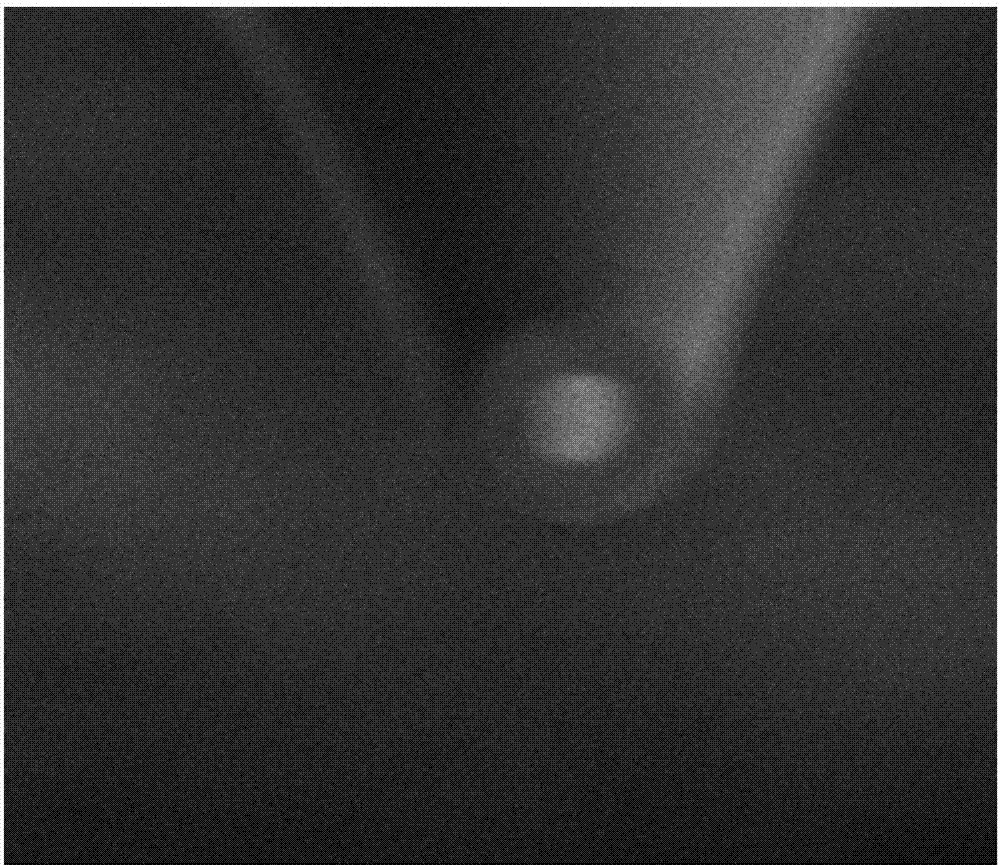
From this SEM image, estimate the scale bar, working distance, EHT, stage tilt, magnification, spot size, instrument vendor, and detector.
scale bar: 1000 nm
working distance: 4.007 mm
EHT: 3 kV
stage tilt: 52°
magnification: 35.1 K X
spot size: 3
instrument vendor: FEI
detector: SED